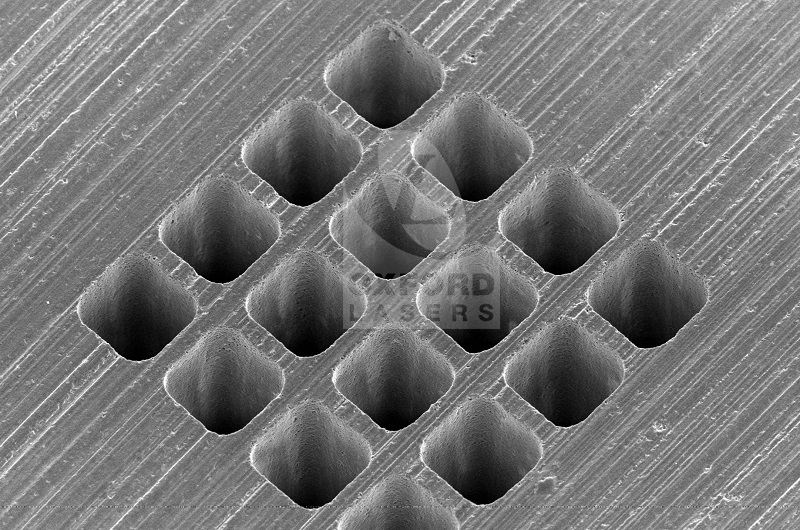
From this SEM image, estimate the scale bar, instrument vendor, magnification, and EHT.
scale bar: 50000 nm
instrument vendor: JEOL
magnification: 0.35 K X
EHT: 10 kV